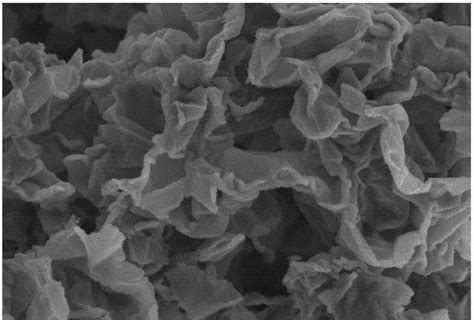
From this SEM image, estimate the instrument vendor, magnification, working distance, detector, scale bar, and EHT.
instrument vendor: Hitachi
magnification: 6 K X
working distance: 9.9 mm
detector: SE(M)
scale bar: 5000 nm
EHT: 5 kV